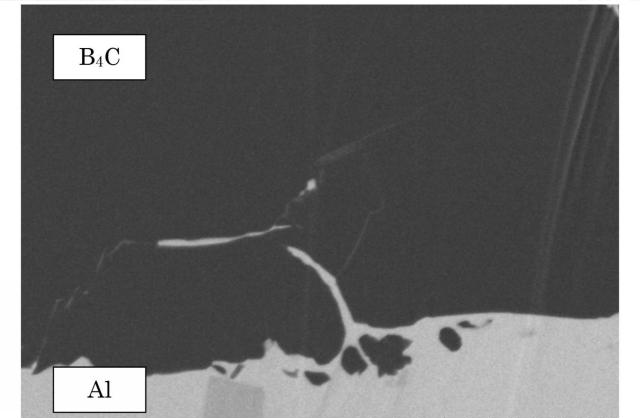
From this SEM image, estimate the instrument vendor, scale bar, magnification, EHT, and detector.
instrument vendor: Hitachi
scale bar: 2000 nm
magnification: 25 K X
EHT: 2 kV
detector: SE(LA30)